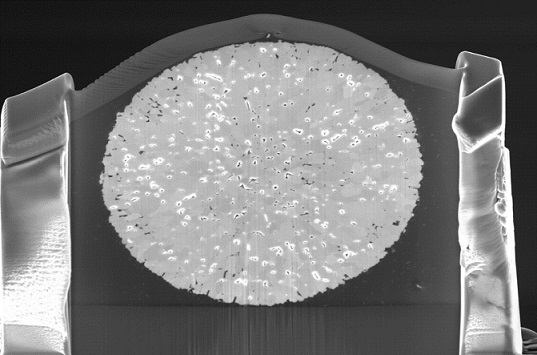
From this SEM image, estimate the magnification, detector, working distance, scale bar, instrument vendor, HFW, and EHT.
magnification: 15 K X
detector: TLD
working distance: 3.9 mm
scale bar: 10000 nm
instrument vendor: FEI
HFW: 27.6 µm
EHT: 5 kV